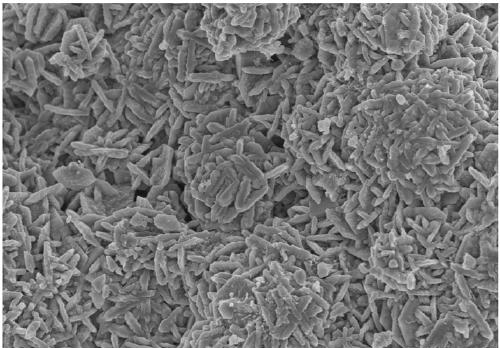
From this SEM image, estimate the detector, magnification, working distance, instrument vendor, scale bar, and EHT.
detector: SE(TUL)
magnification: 6 K X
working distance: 7.2 mm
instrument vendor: Hitachi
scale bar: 5000 nm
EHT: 5 kV